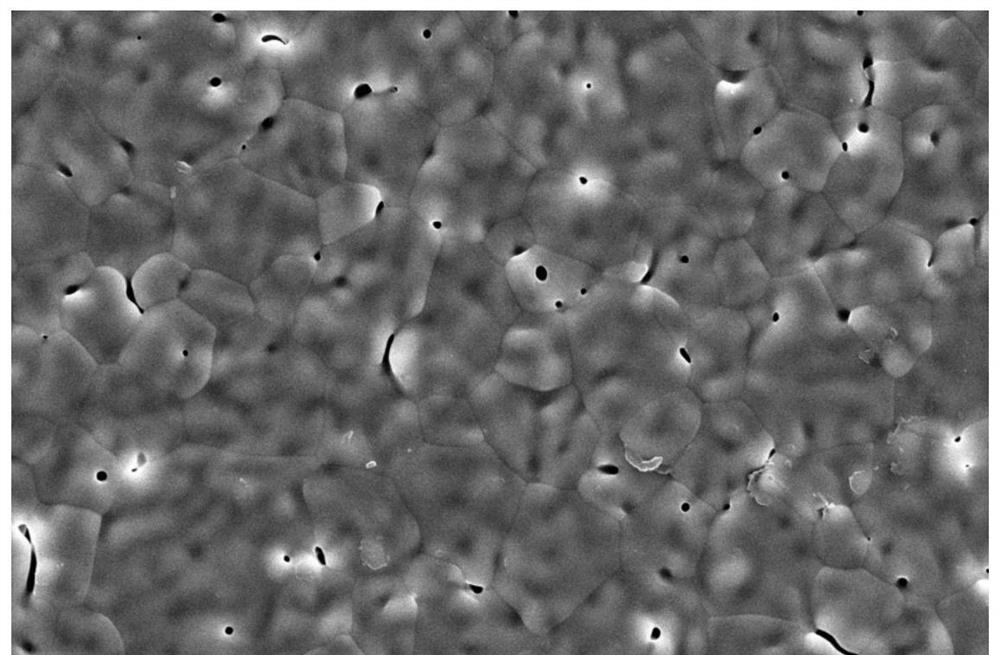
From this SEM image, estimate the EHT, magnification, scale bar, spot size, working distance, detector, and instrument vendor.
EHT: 20 kV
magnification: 10 K X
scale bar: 10000 nm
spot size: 8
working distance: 9.5 mm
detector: ETD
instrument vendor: FEI